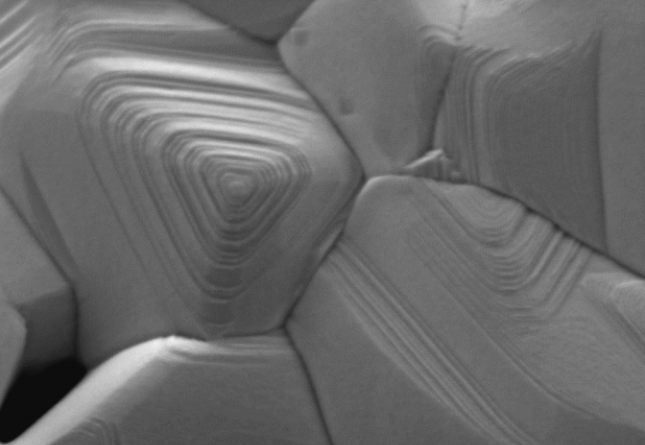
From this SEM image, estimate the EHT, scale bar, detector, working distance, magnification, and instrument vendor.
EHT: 1 kV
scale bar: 100 nm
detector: InLens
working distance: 0.9 mm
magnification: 150 K X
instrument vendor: Zeiss